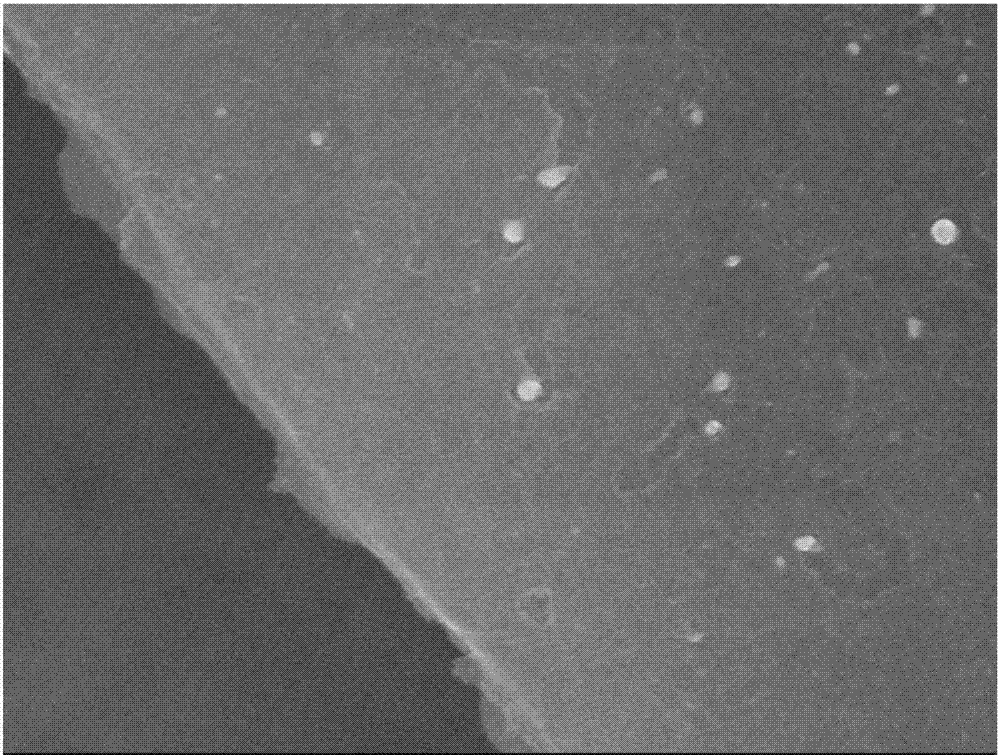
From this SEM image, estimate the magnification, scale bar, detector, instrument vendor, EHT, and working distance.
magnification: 30 K X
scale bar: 100 nm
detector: SEI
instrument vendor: JEOL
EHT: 10 kV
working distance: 8.6 mm